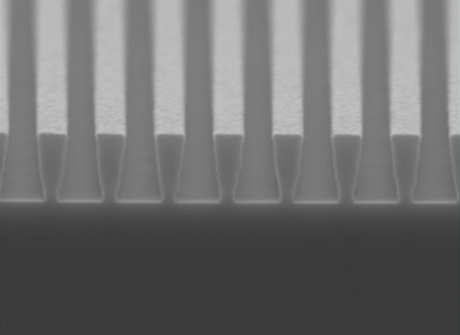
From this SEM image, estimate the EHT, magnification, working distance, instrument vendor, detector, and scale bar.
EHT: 5 kV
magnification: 40 K X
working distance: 9.9 mm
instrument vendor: JEOL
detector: LED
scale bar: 100 nm